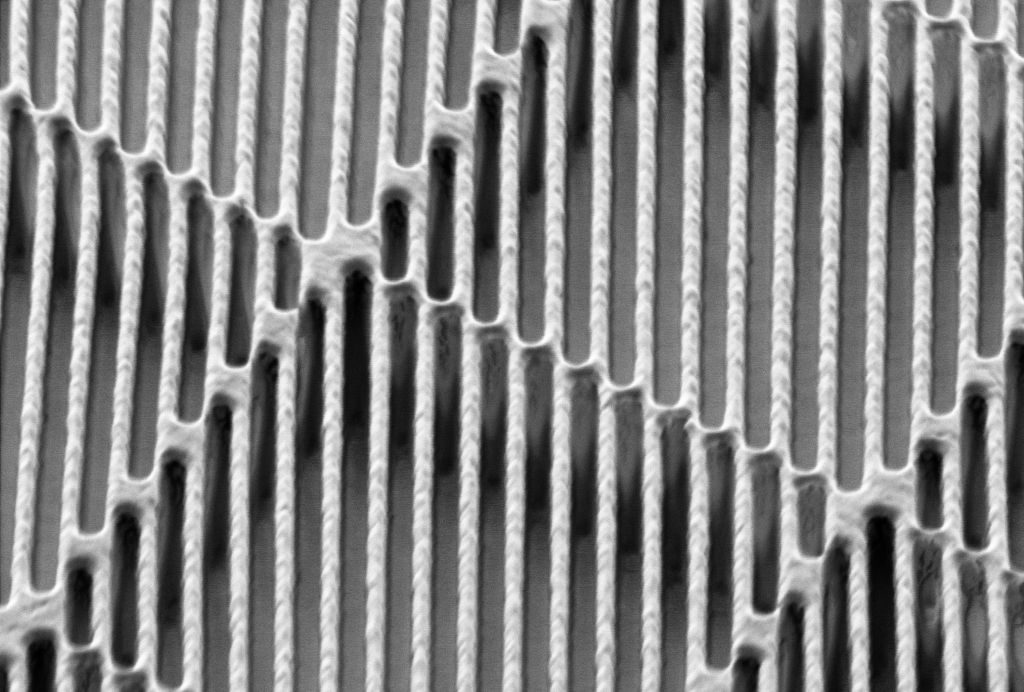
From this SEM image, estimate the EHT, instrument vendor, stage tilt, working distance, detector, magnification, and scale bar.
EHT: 2 kV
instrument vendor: Zeiss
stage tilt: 54°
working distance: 5 mm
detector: SESI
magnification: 16.93 K X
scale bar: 200 nm